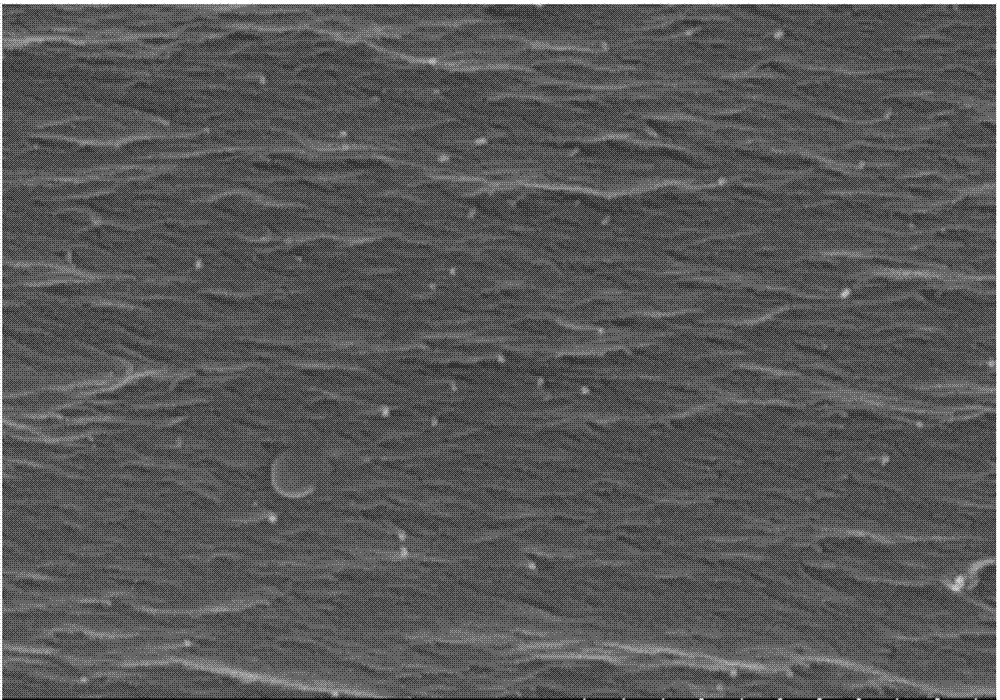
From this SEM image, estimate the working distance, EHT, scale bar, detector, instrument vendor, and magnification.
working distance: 14.8 mm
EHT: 15 kV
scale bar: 1000 nm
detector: SE(U)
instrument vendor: Hitachi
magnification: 50 K X